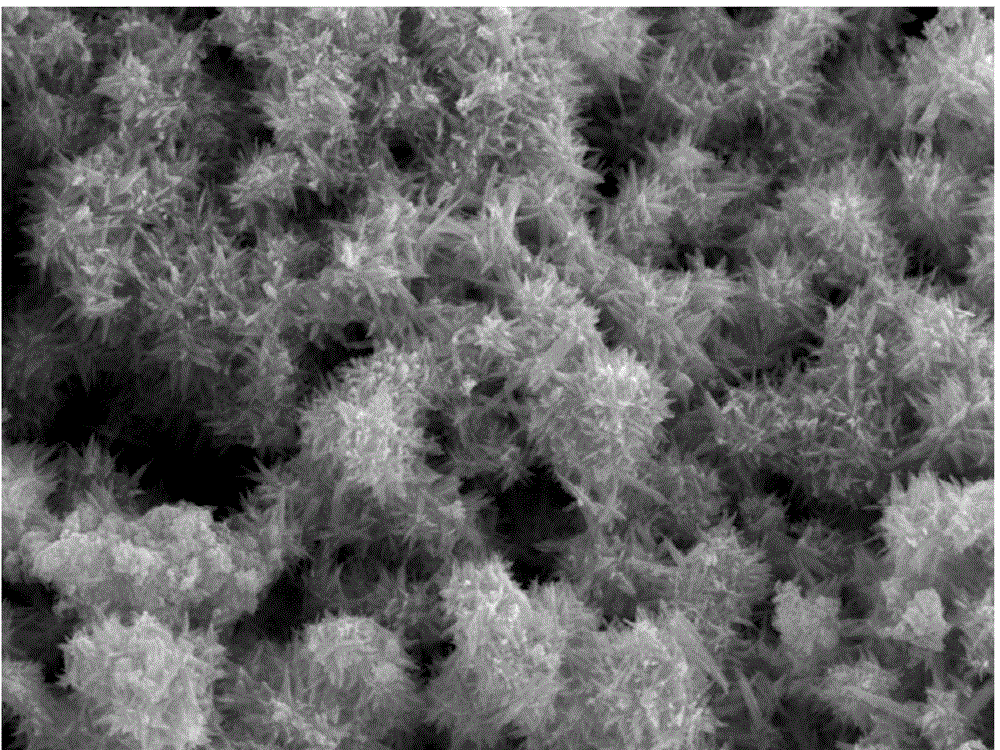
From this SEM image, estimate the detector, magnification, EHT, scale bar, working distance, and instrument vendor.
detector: SEI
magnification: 20 K X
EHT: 10 kV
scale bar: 1000 nm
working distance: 10 mm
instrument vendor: JEOL